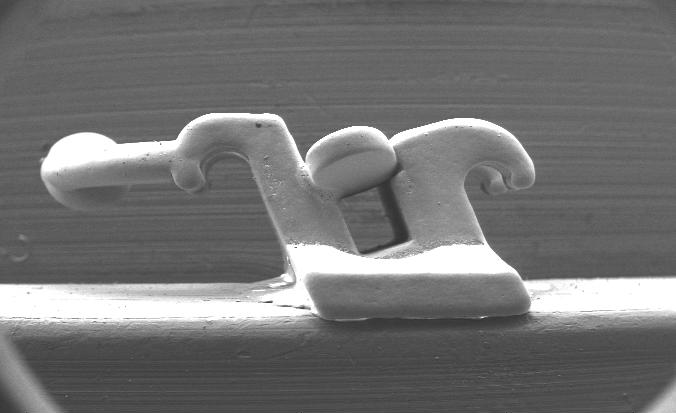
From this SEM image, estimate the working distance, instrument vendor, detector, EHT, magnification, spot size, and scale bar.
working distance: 13.4 mm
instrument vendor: FEI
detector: SE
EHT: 20 kV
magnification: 0.035 K X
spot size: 7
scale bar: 2000000 nm